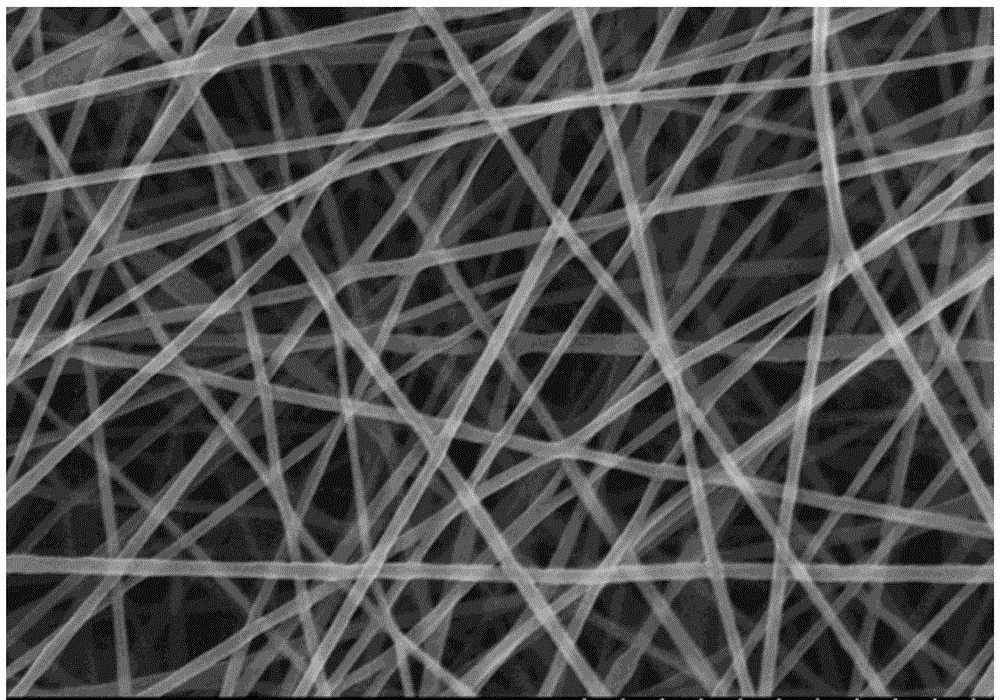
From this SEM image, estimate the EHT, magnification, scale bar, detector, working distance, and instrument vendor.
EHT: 15 kV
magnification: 10 K X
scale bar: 5000 nm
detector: SE(M)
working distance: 6.3 mm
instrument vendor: Hitachi